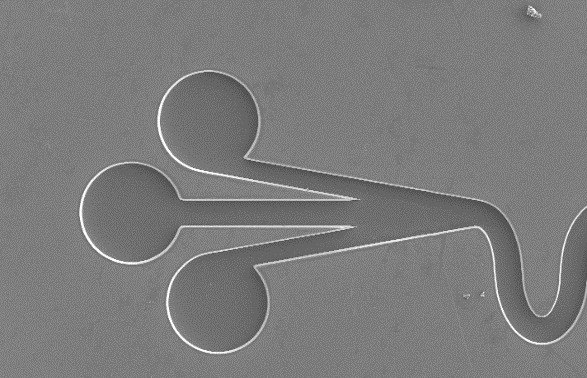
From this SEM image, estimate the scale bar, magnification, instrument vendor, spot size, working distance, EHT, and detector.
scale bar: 100000 nm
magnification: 0.275 K X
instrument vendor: FEI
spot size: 3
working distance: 7 mm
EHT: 5 kV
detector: SE